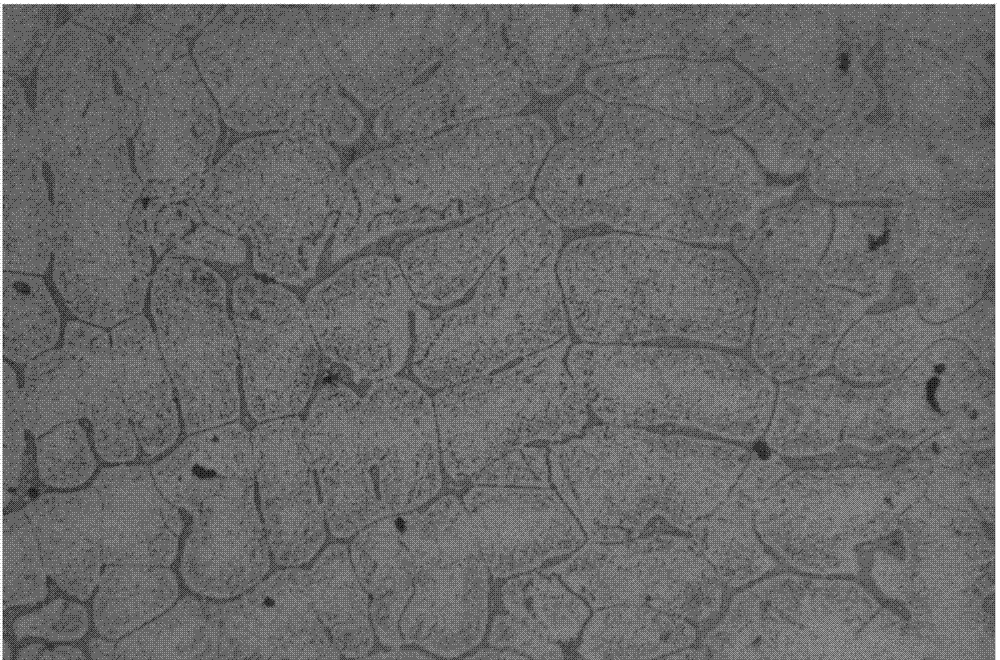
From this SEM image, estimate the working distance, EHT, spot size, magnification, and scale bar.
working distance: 7.9 mm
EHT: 2 kV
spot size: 3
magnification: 10 K X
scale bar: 1000 nm